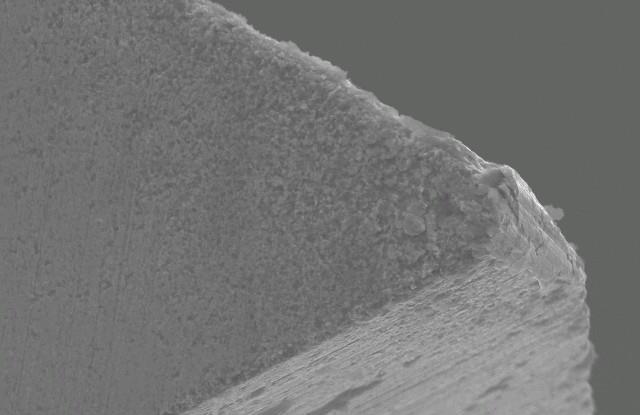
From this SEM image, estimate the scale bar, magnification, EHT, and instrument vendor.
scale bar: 10000 nm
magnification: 1 K X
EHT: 20 kV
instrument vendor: JEOL